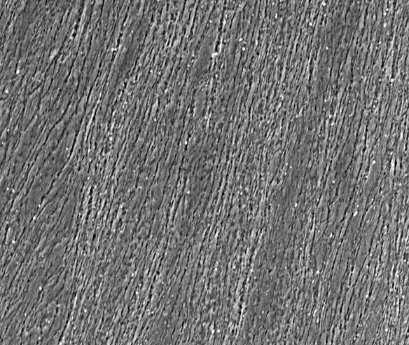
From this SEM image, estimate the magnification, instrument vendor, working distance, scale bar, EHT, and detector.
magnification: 4 K X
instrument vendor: FEI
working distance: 8 mm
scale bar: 40000 nm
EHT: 20 kV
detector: ETD